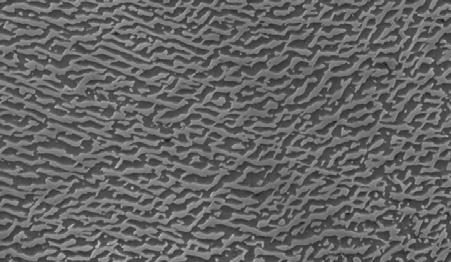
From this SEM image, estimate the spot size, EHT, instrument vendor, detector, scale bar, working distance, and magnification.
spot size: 4.1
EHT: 20 kV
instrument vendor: FEI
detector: SE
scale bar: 10000 nm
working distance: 9.4 mm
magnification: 2.5 K X